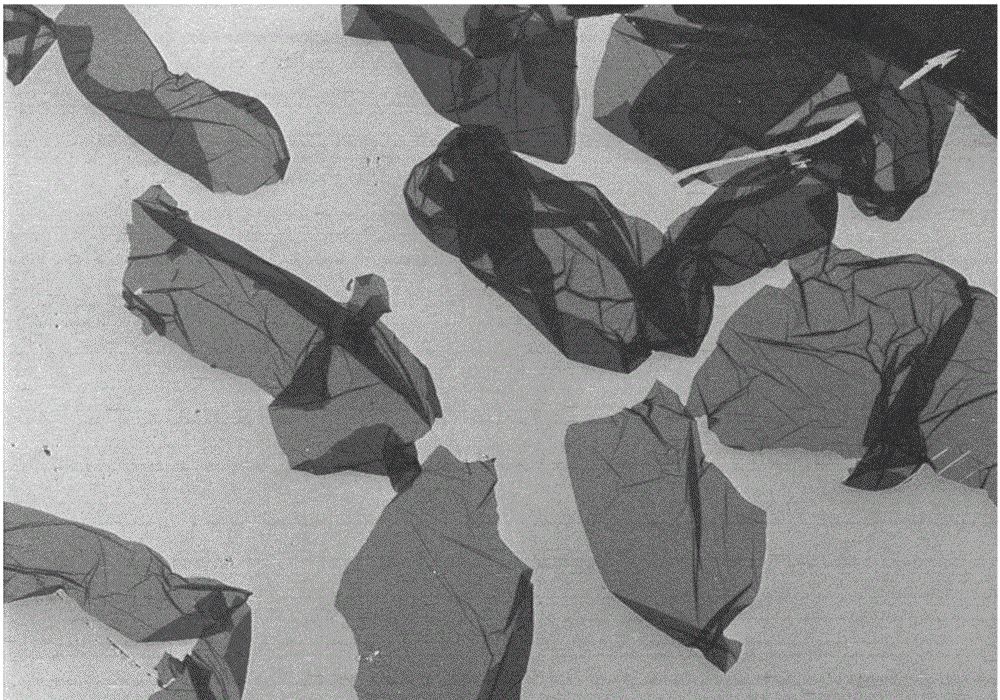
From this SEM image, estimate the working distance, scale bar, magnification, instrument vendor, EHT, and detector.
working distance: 8.5 mm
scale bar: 50000 nm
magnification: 0.6 K X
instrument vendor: Hitachi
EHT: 3 kV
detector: SE(M)